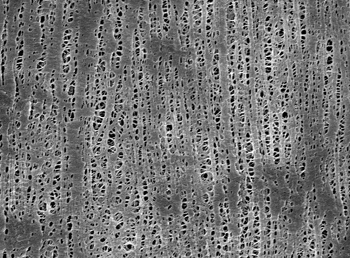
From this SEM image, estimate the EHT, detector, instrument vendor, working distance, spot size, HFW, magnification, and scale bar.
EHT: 5 kV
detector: TLD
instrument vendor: FEI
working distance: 5.6 mm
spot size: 3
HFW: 10.3 µm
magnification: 12 K X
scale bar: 2000 nm